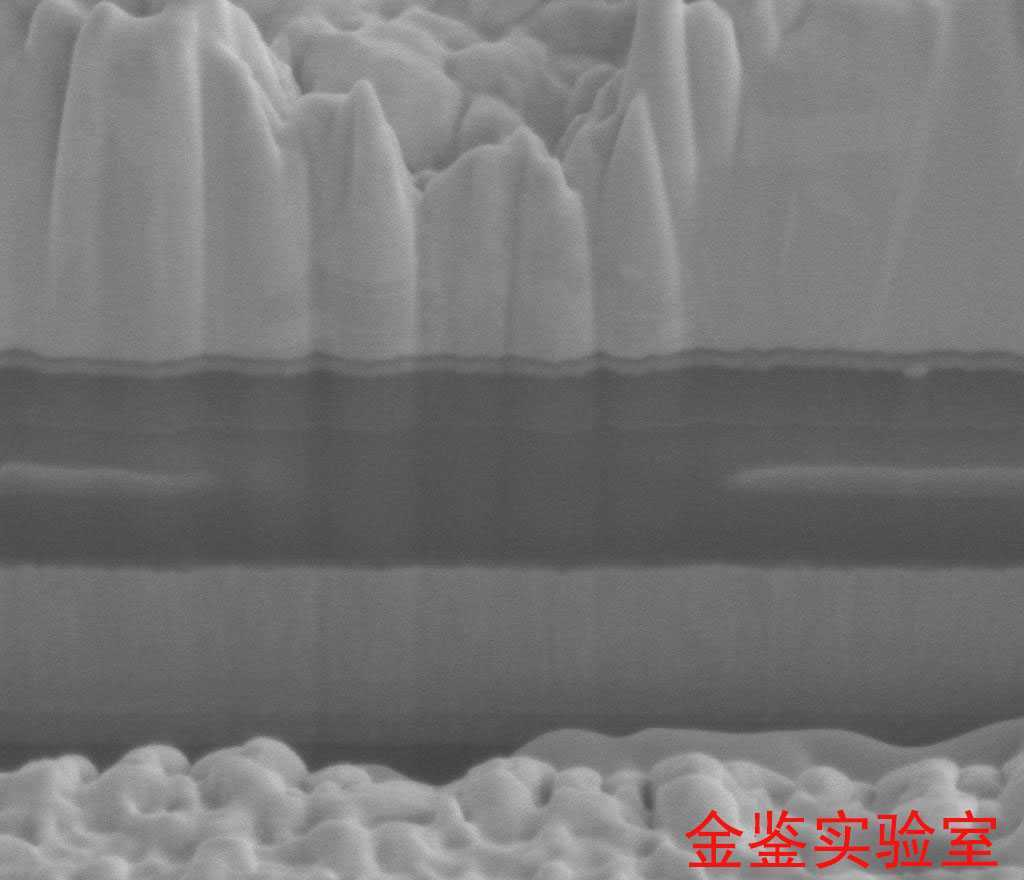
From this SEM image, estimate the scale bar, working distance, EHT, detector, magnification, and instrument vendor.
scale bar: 1000 nm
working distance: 4.9 mm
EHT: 5 kV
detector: ETD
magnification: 60 K X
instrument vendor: FEI